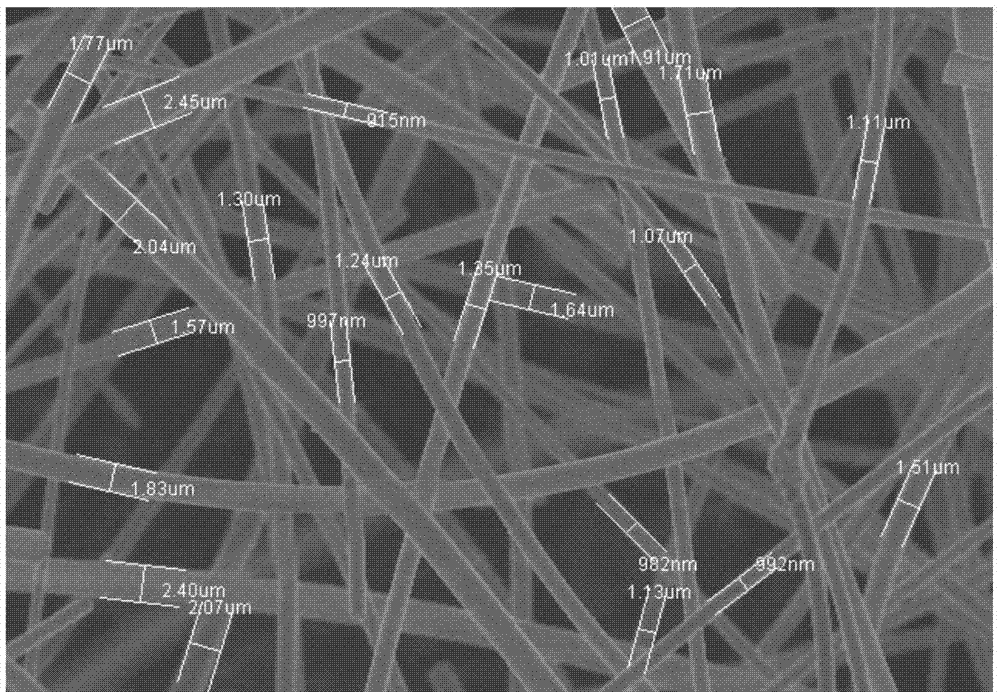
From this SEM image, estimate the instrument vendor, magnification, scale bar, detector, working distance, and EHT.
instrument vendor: Hitachi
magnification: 2 K X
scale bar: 20000 nm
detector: SE(M)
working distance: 8.5 mm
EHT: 5 kV